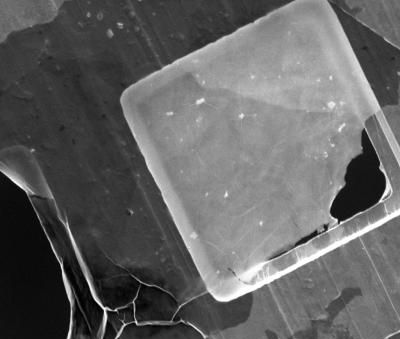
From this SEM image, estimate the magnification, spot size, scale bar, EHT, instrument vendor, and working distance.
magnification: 2 K X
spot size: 3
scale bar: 40000 nm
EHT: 5 kV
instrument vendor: FEI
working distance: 10.7 mm